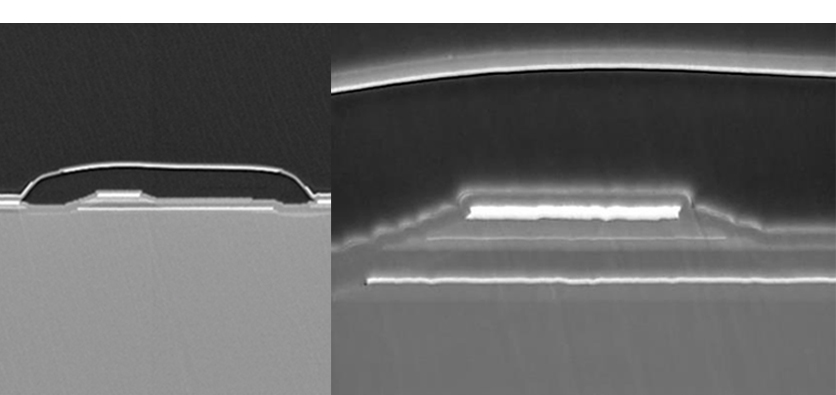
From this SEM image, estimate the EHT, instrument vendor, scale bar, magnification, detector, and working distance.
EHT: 2 kV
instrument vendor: JEOL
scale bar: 1000 nm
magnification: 20 K X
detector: BED-C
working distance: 10 mm